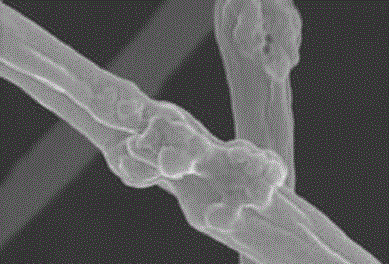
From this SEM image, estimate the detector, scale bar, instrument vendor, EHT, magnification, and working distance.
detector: SE(M)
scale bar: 1000 nm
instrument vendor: Hitachi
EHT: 5 kV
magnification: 45 K X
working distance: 9.3 mm